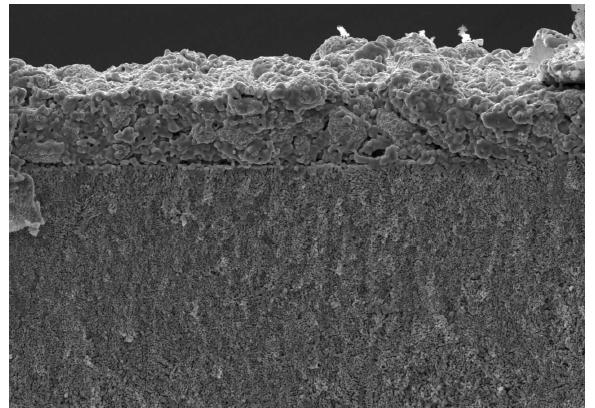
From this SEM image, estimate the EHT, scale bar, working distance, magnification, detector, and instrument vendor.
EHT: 15 kV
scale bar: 50000 nm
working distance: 12.1 mm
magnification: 1 K X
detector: SE(U)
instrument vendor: Hitachi